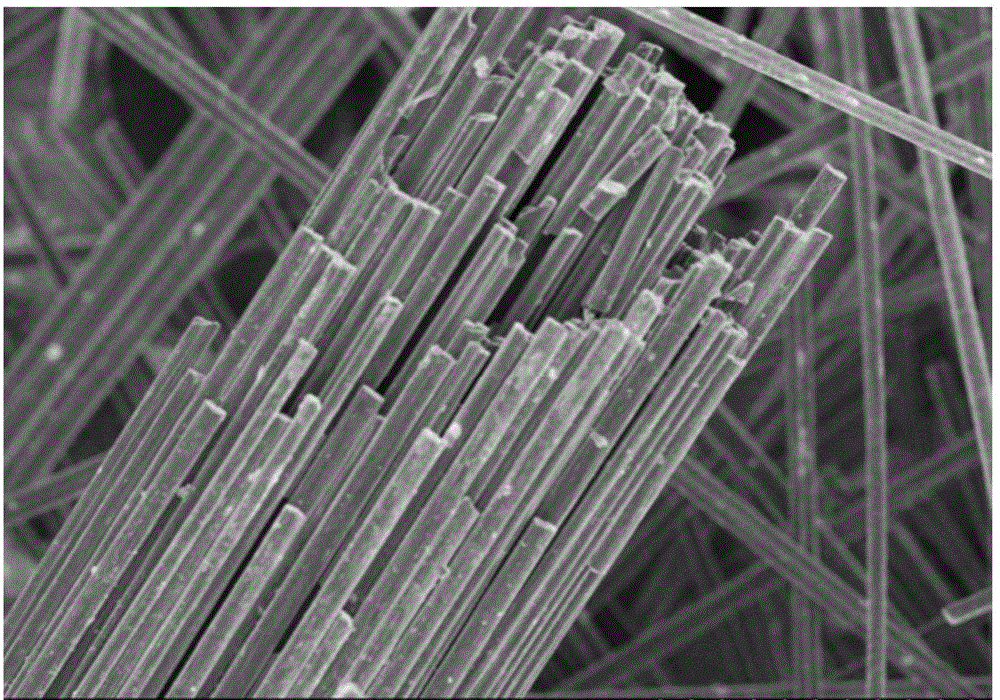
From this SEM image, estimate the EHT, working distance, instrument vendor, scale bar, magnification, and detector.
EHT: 5 kV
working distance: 8.3 mm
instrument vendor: Hitachi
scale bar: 100000 nm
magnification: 0.4 K X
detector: SE(M)